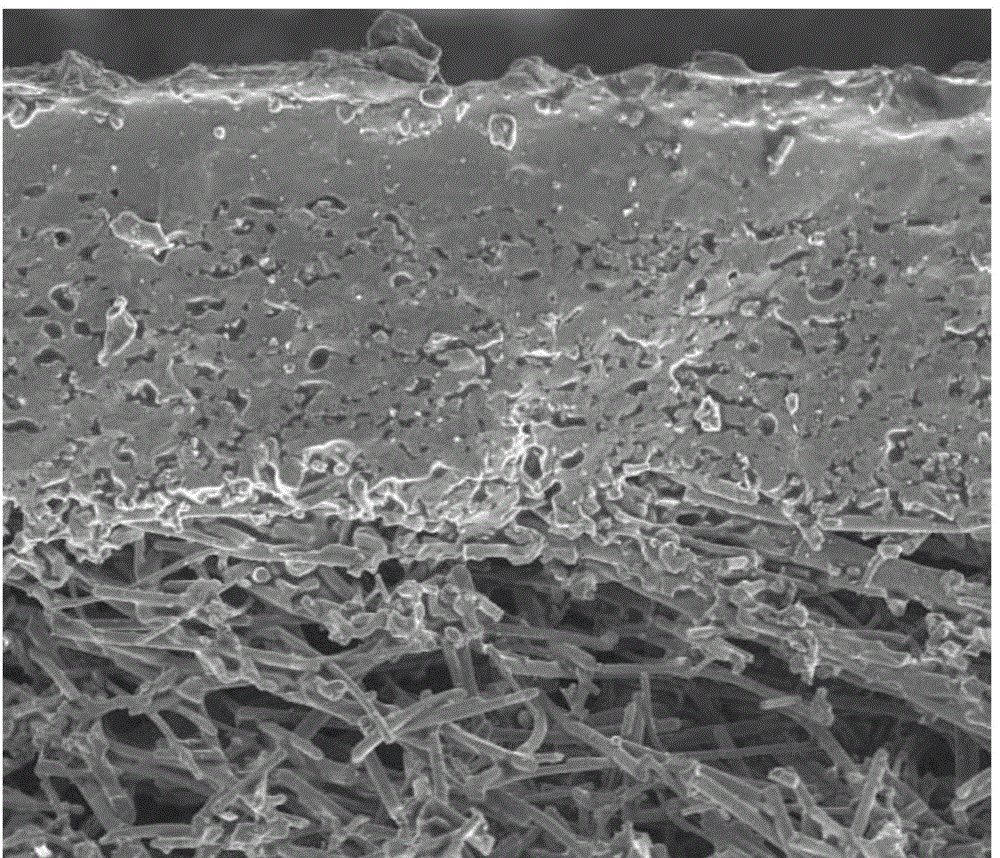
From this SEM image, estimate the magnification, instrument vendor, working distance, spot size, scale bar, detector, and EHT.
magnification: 0.3 K X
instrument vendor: FEI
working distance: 10 mm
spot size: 5.5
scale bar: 100000 nm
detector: ETD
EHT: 12.5 kV